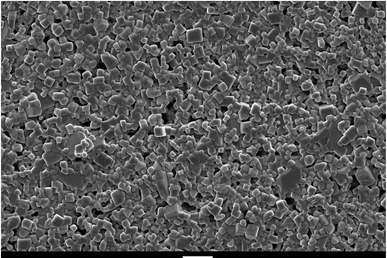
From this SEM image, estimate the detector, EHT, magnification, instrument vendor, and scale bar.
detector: SEI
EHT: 15 kV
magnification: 1 K X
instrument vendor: JEOL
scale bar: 10000 nm